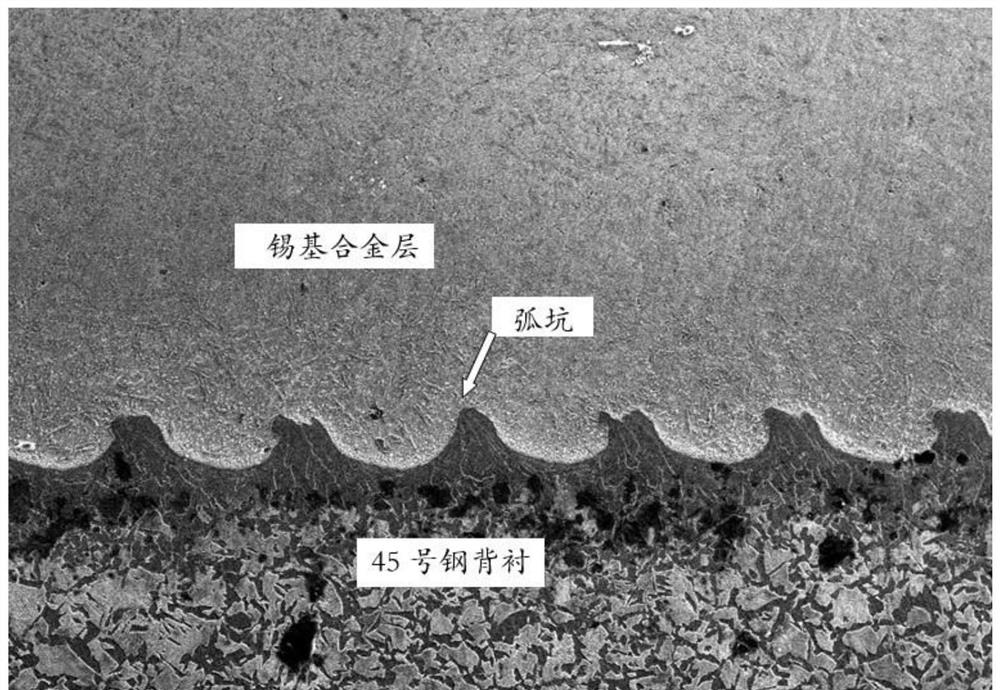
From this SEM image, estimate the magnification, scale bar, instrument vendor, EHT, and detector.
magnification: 0.1 K X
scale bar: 100000 nm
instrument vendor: JEOL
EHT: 20 kV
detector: SEI